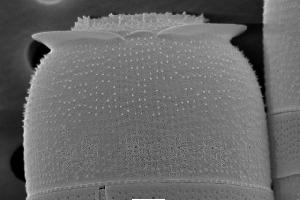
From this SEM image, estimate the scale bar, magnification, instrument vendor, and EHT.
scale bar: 2000 nm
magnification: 7 K X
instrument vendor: JEOL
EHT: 10 kV